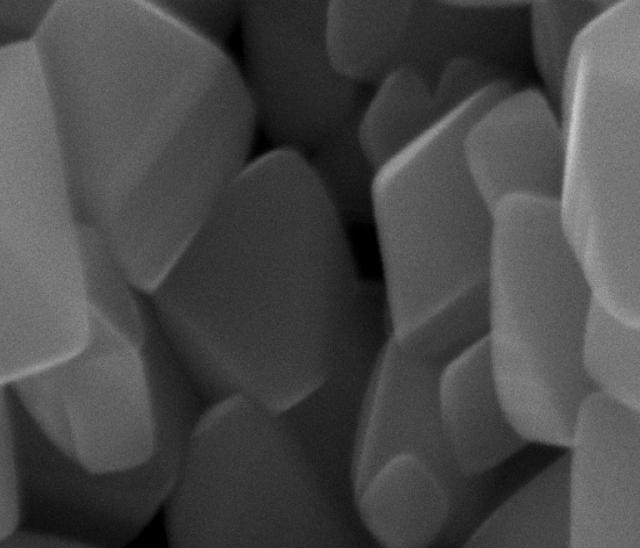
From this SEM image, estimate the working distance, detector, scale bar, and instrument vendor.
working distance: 4.4 mm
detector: ETD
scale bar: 500 nm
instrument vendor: FEI